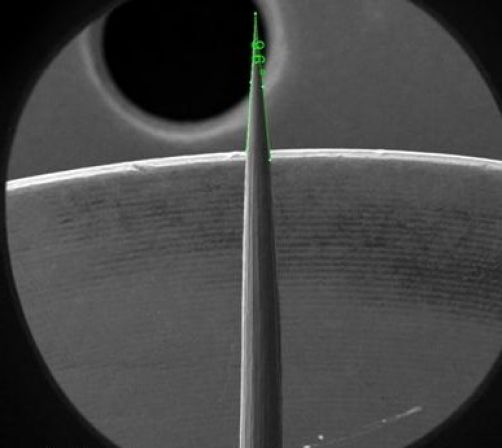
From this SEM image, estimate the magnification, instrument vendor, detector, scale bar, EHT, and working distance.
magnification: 0.06 K X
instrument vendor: FEI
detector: ETD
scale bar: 1000000 nm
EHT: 2 kV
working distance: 5.3 mm